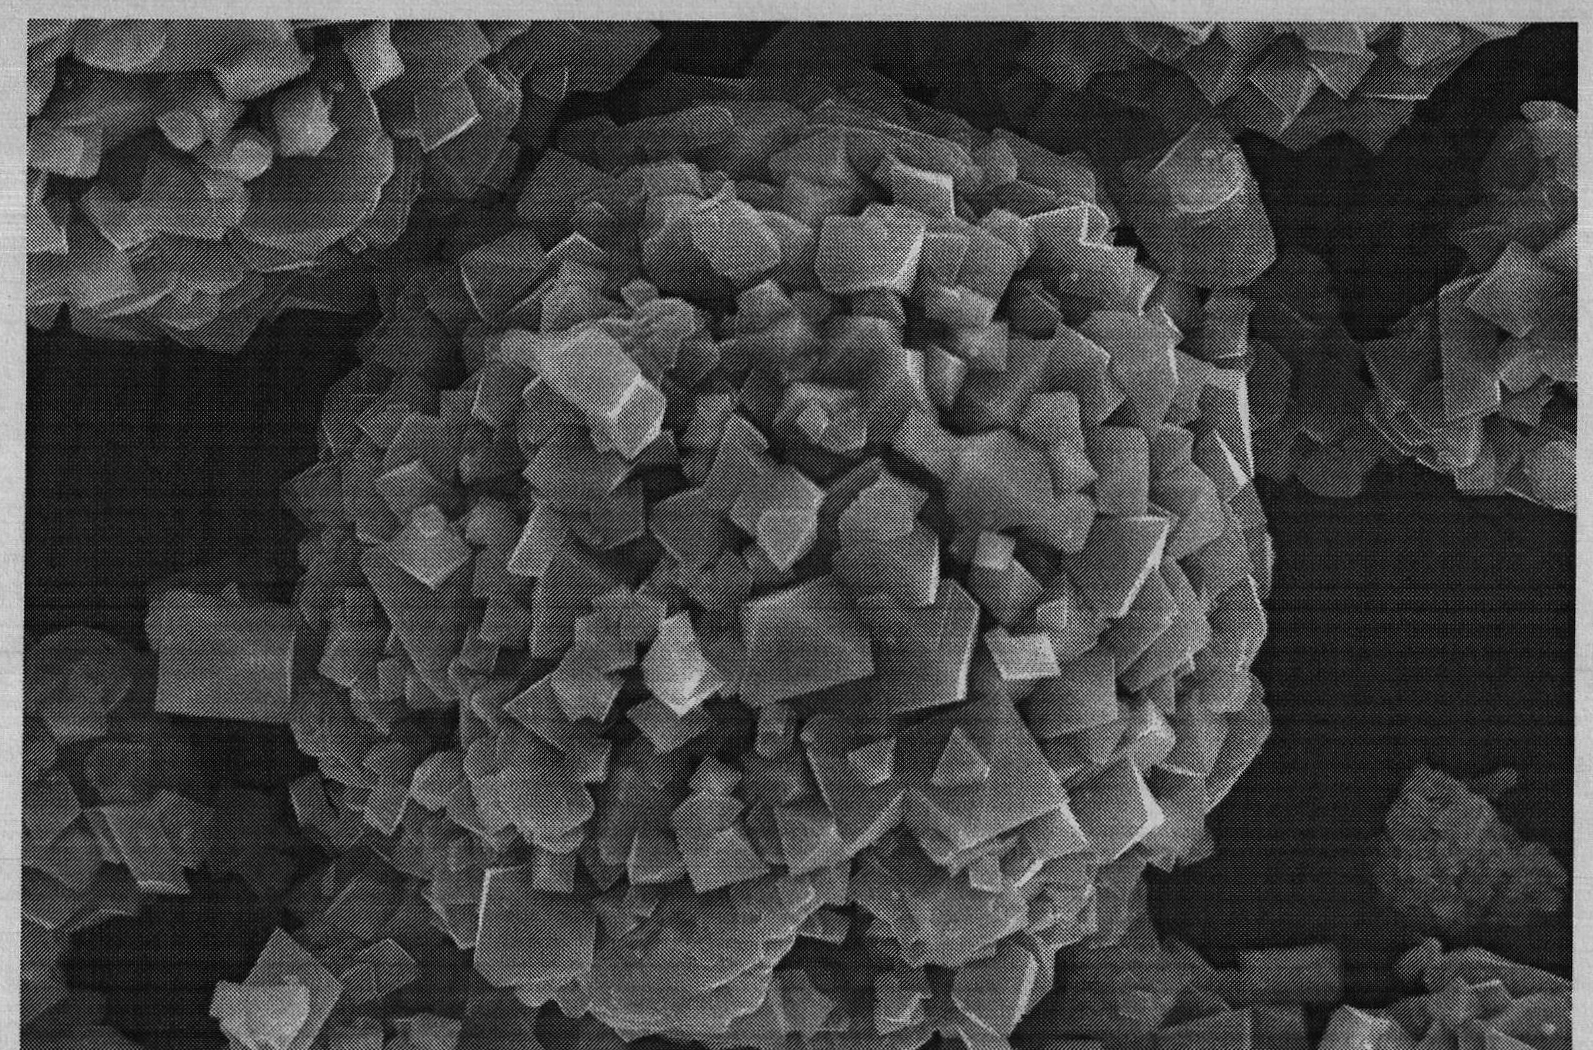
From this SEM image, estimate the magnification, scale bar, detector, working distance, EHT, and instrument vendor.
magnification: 3 K X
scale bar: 5000 nm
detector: SEI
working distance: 10 mm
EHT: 20 kV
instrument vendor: JEOL